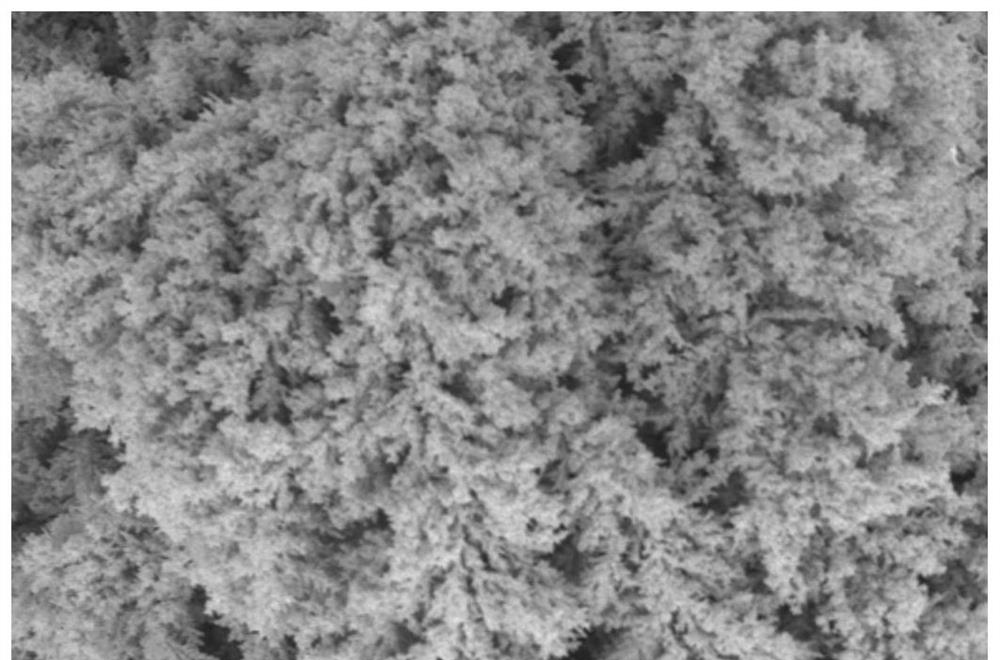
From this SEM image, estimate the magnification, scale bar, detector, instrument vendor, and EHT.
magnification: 2 K X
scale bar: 10000 nm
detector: SEI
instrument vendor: JEOL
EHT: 15 kV